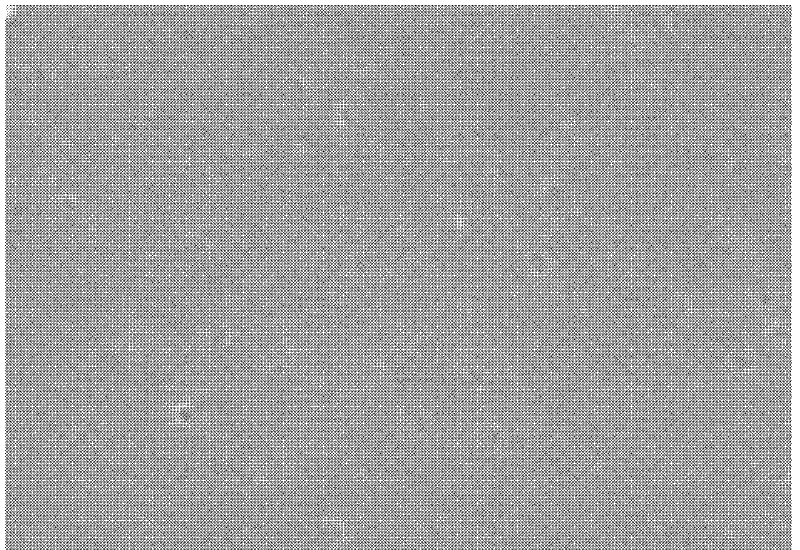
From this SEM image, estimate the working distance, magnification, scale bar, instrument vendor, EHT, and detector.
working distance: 14.7 mm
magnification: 130 K X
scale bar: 400 nm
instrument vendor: Hitachi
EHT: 20 kV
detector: SE(U)